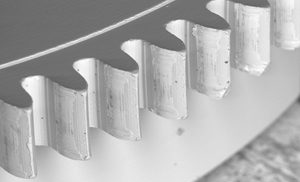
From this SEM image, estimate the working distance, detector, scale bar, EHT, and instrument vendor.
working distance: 21 mm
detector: BSE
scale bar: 600000 nm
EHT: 20 kV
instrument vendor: Tescan